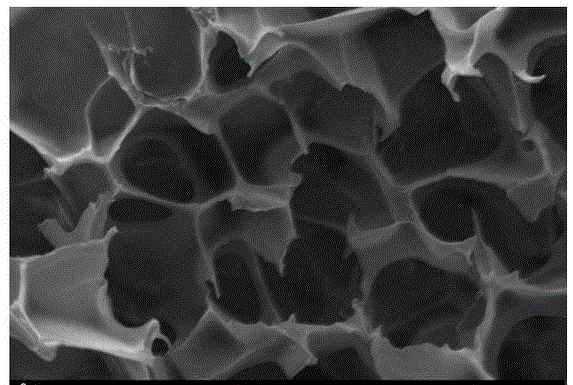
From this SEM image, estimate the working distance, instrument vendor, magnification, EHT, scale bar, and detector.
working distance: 6.5 mm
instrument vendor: Zeiss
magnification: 2 K X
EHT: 10 kV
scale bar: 2000 nm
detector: SE1